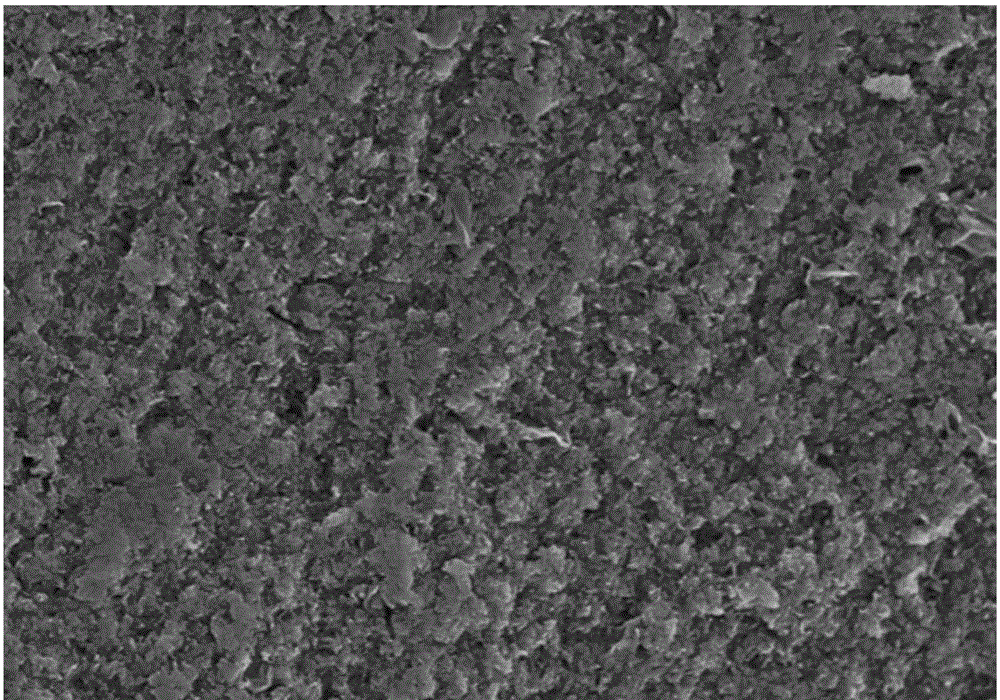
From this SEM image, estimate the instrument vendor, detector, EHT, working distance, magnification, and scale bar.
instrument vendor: Hitachi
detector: SE(M)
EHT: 5 kV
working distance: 9.7 mm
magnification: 5 K X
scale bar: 10000 nm